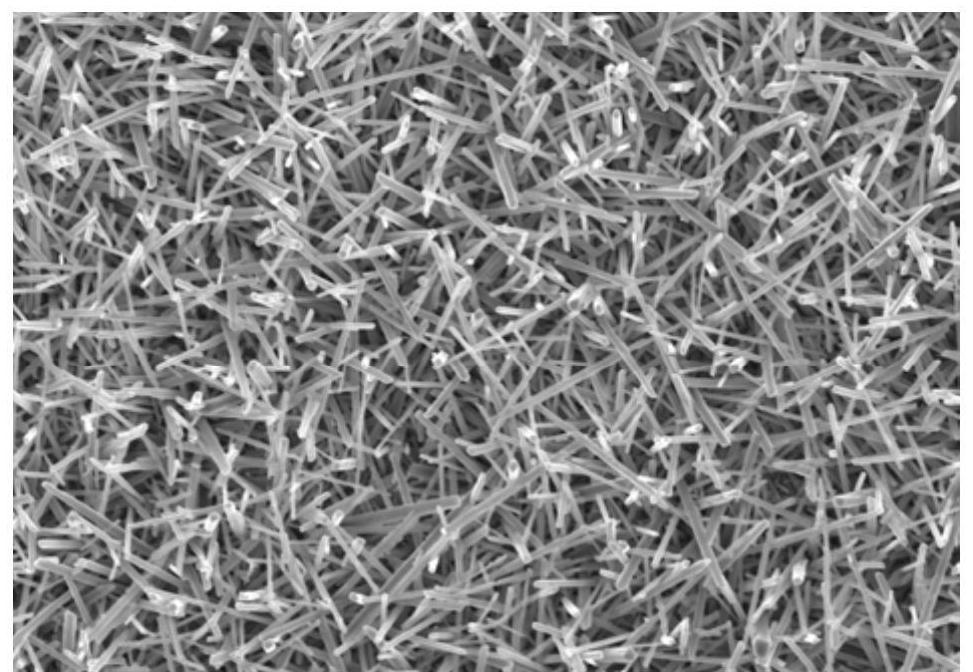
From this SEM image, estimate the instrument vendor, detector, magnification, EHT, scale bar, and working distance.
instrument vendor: Hitachi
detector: SE(UL)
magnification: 5 K X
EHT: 10 kV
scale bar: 20000 nm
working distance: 11.6 mm